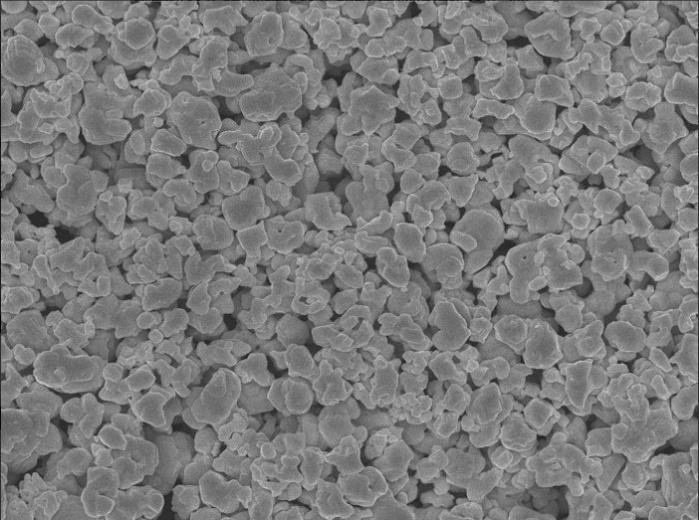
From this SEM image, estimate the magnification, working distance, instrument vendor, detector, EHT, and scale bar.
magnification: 10 K X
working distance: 8 mm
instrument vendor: JEOL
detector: SEI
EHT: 10 kV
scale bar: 1000 nm